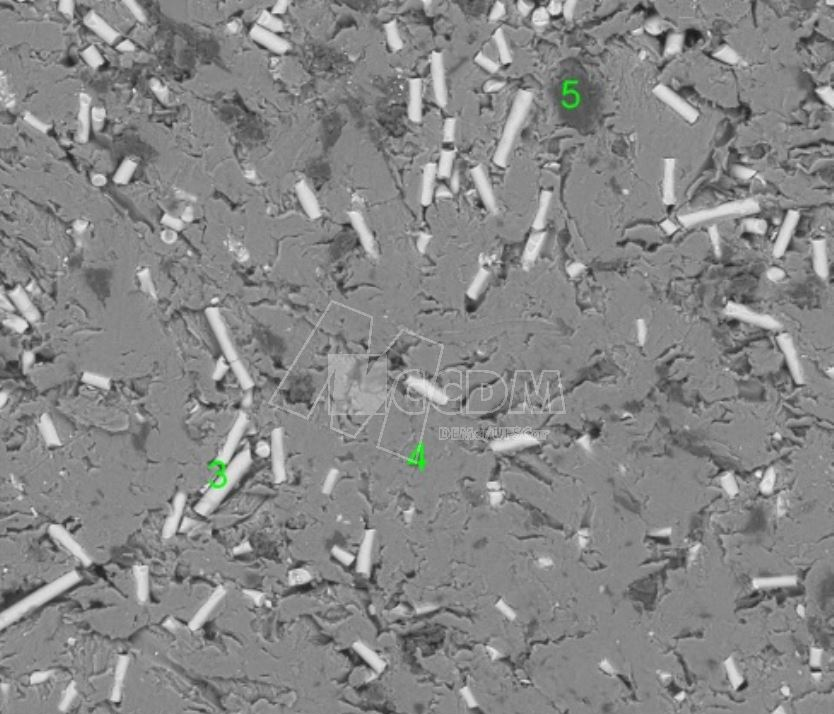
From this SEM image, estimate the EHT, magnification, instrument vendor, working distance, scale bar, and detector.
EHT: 20 kV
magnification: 0.3 K X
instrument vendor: FEI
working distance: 10.5 mm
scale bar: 300000 nm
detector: BSED